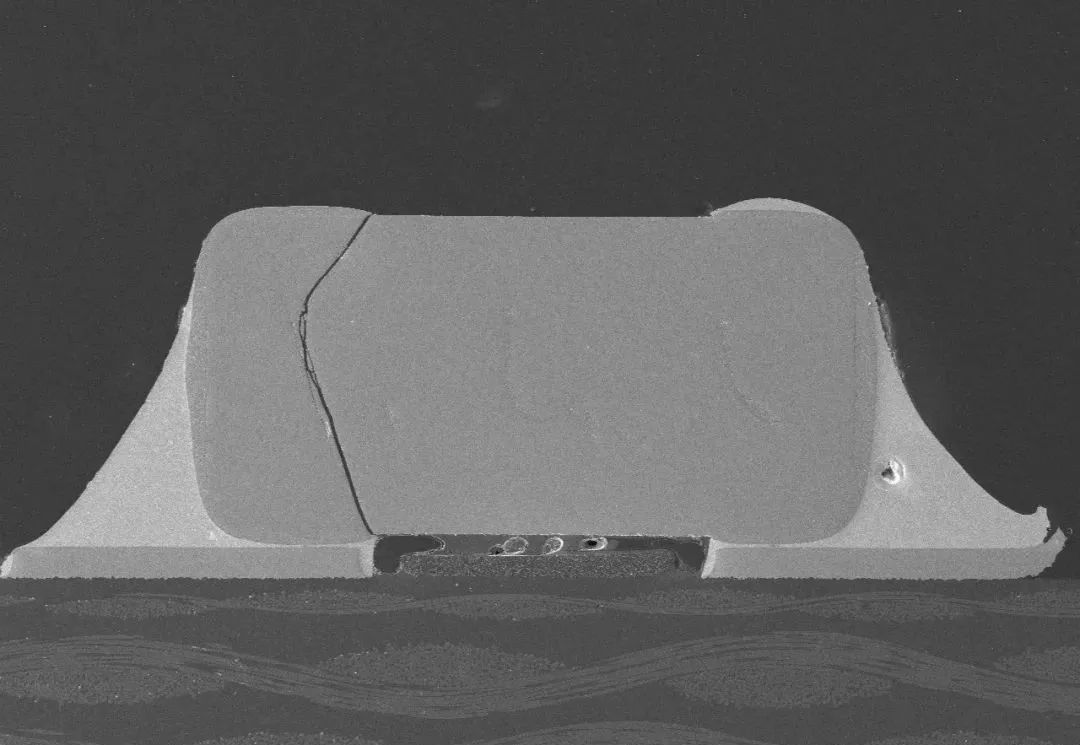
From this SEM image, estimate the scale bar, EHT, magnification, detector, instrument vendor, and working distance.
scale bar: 1000000 nm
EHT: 10 kV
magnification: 0.05 K X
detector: LM(L)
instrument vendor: Hitachi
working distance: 15.4 mm